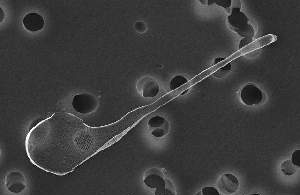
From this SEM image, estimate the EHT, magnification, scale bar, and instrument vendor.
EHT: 10 kV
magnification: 3 K X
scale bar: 5000 nm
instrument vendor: JEOL